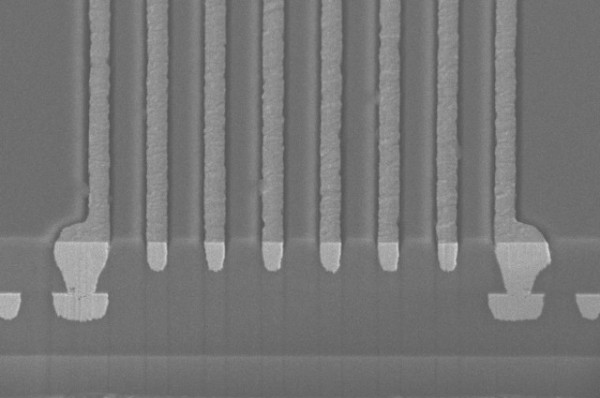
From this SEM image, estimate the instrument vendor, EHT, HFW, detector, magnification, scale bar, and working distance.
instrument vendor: FEI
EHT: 15 kV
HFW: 104 µm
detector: TLD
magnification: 2 K X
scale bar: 30000 nm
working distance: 4 mm